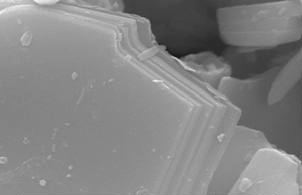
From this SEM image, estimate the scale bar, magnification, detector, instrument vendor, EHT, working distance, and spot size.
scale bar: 1000 nm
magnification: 30 K X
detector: SE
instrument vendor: FEI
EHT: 15 kV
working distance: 10 mm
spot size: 3